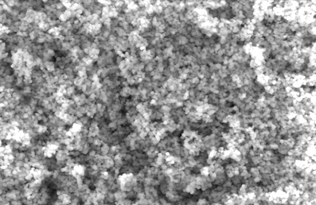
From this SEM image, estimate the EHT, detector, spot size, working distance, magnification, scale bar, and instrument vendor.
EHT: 25 kV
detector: SE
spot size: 5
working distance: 9.5 mm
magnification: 5 K X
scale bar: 5000 nm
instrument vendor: FEI